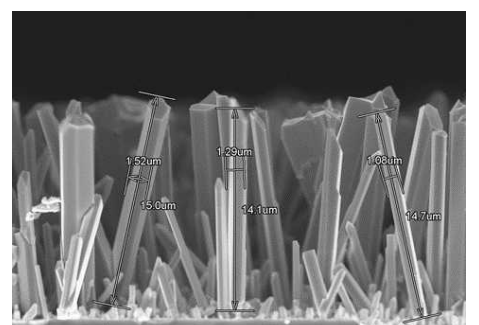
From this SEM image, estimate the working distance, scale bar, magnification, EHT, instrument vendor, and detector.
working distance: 7.7 mm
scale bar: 10000 nm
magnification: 4 K X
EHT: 10 kV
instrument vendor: Hitachi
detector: SE(M)